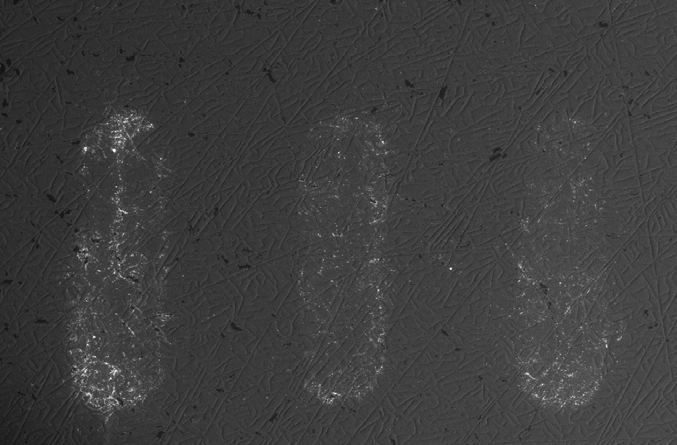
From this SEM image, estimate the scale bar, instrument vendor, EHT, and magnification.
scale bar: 1000000 nm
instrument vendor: JEOL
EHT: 15 kV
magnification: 0.023 K X